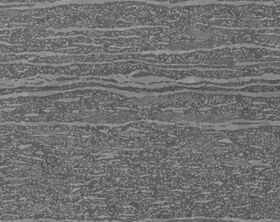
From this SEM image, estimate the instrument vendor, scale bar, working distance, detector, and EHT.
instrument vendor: Zeiss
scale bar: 10000 nm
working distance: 3.8 mm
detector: InLens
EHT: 5 kV